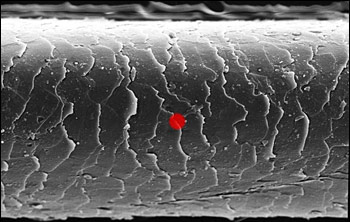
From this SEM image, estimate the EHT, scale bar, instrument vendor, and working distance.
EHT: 10 kV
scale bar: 10000 nm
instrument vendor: JEOL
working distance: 15 mm